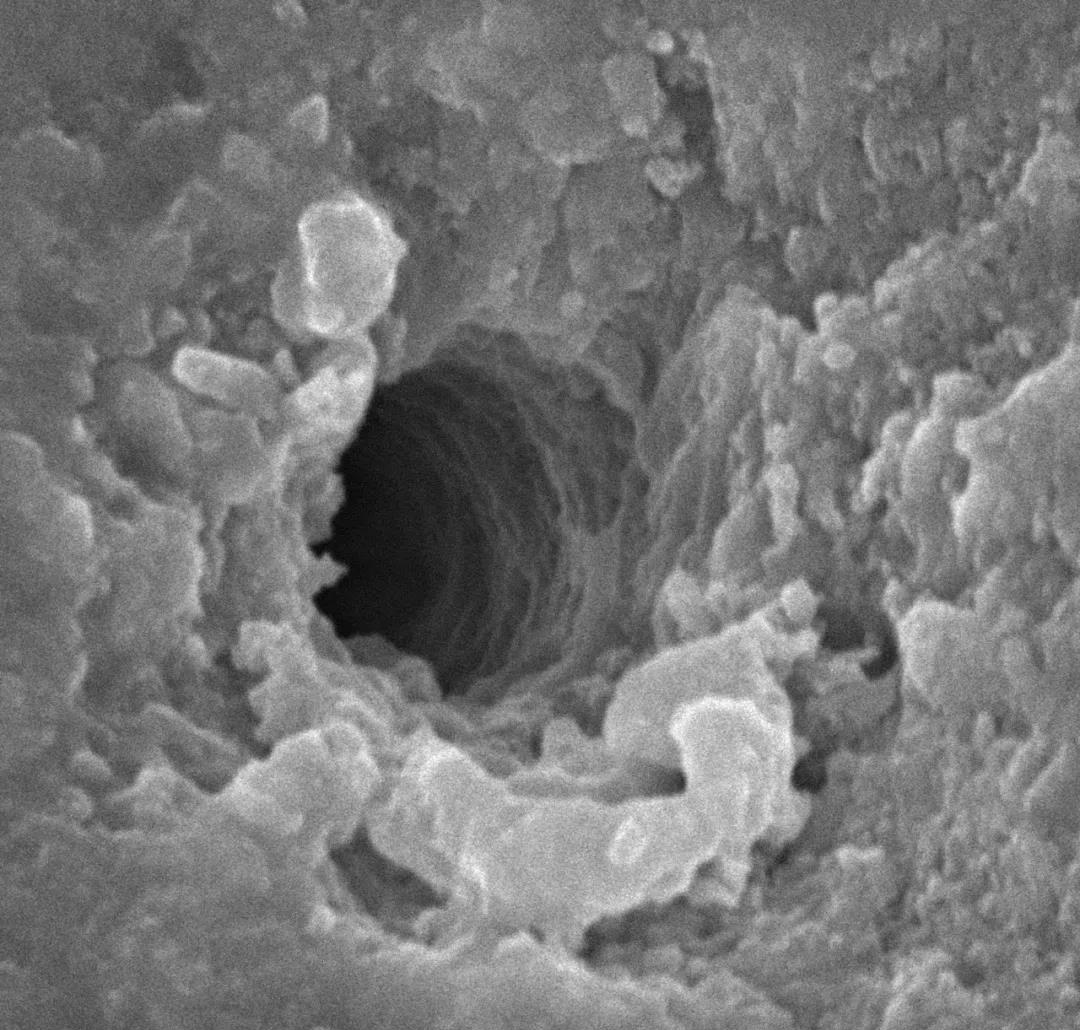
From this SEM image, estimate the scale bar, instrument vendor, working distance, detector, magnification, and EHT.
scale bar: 2000 nm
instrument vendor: other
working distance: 8.9 mm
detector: SE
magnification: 20 K X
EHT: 15 kV